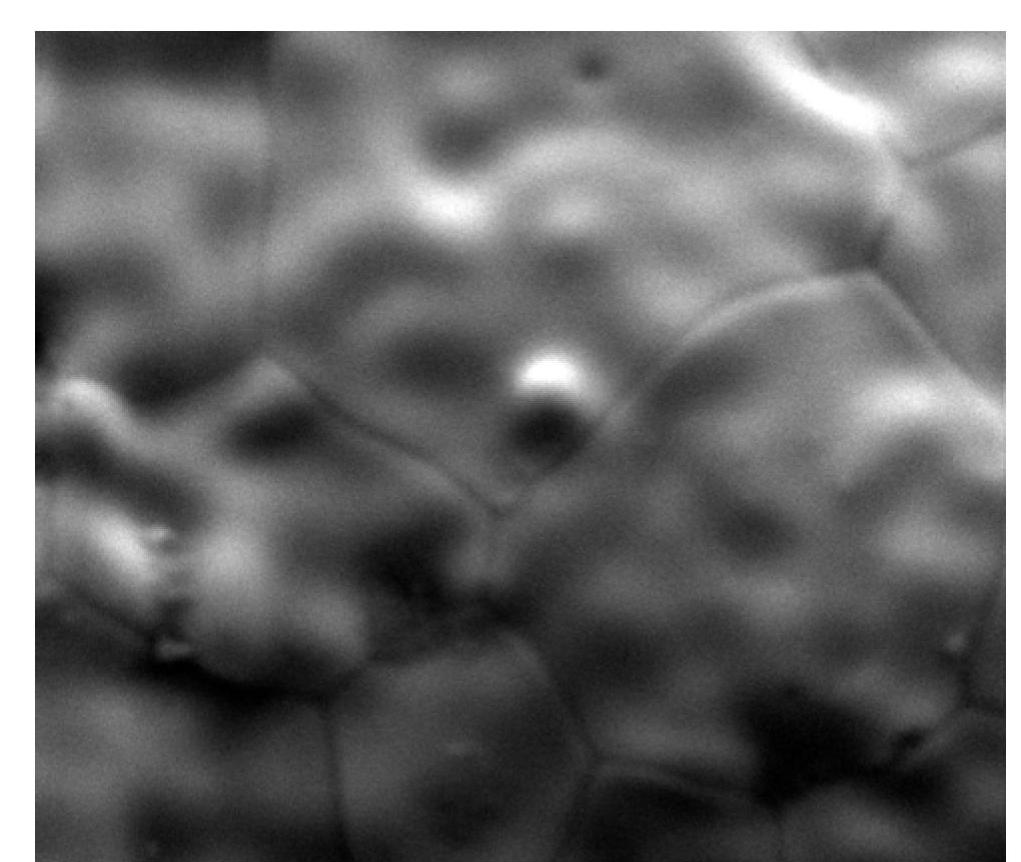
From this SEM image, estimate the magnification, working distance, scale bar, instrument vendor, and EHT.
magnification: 2.5 K X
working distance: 13.1 mm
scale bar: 20000 nm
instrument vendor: FEI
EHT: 20 kV